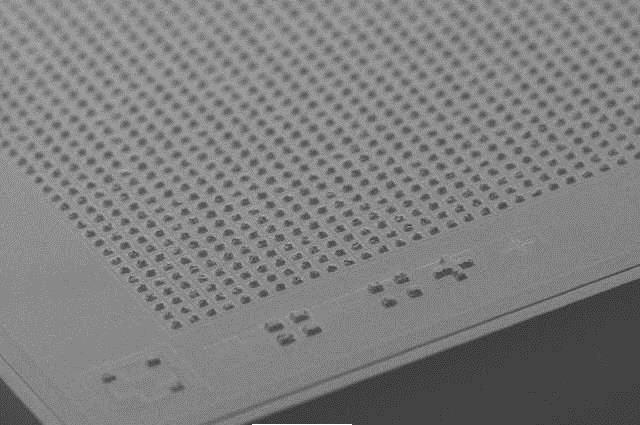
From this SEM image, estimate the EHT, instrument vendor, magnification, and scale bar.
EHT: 20 kV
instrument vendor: JEOL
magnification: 0.2 K X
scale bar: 100000 nm